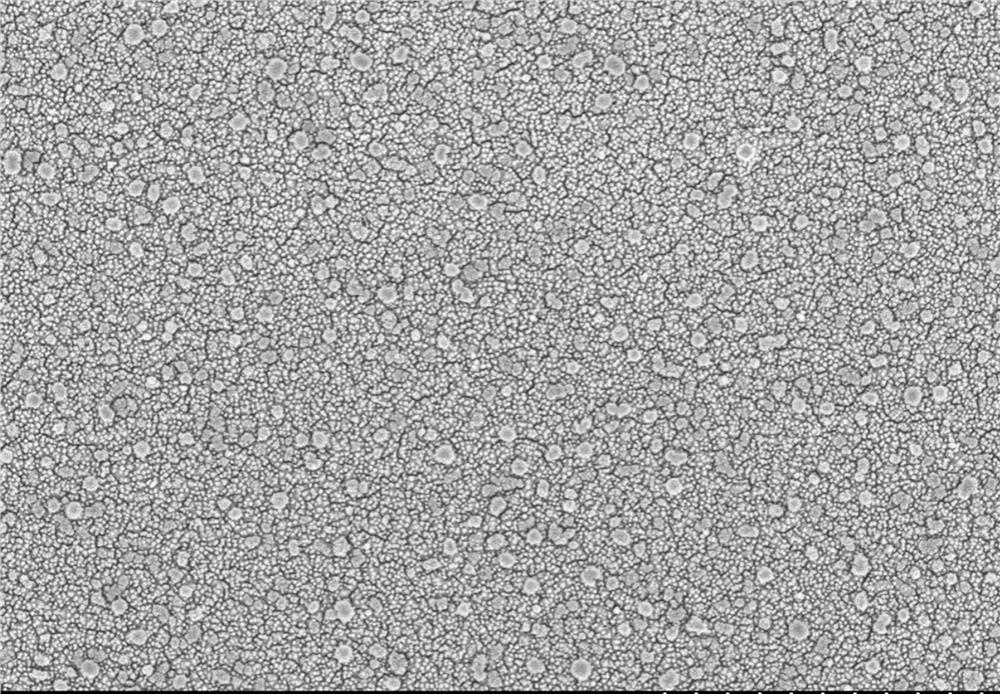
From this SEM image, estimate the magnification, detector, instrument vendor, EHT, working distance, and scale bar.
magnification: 20 K X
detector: SE(M)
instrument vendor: Hitachi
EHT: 3 kV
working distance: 8.8 mm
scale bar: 2000 nm